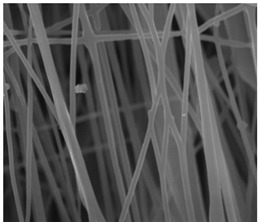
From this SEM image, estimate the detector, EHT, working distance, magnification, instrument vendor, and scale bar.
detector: SEI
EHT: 15 kV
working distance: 8 mm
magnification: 6 K X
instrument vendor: JEOL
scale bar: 1000 nm